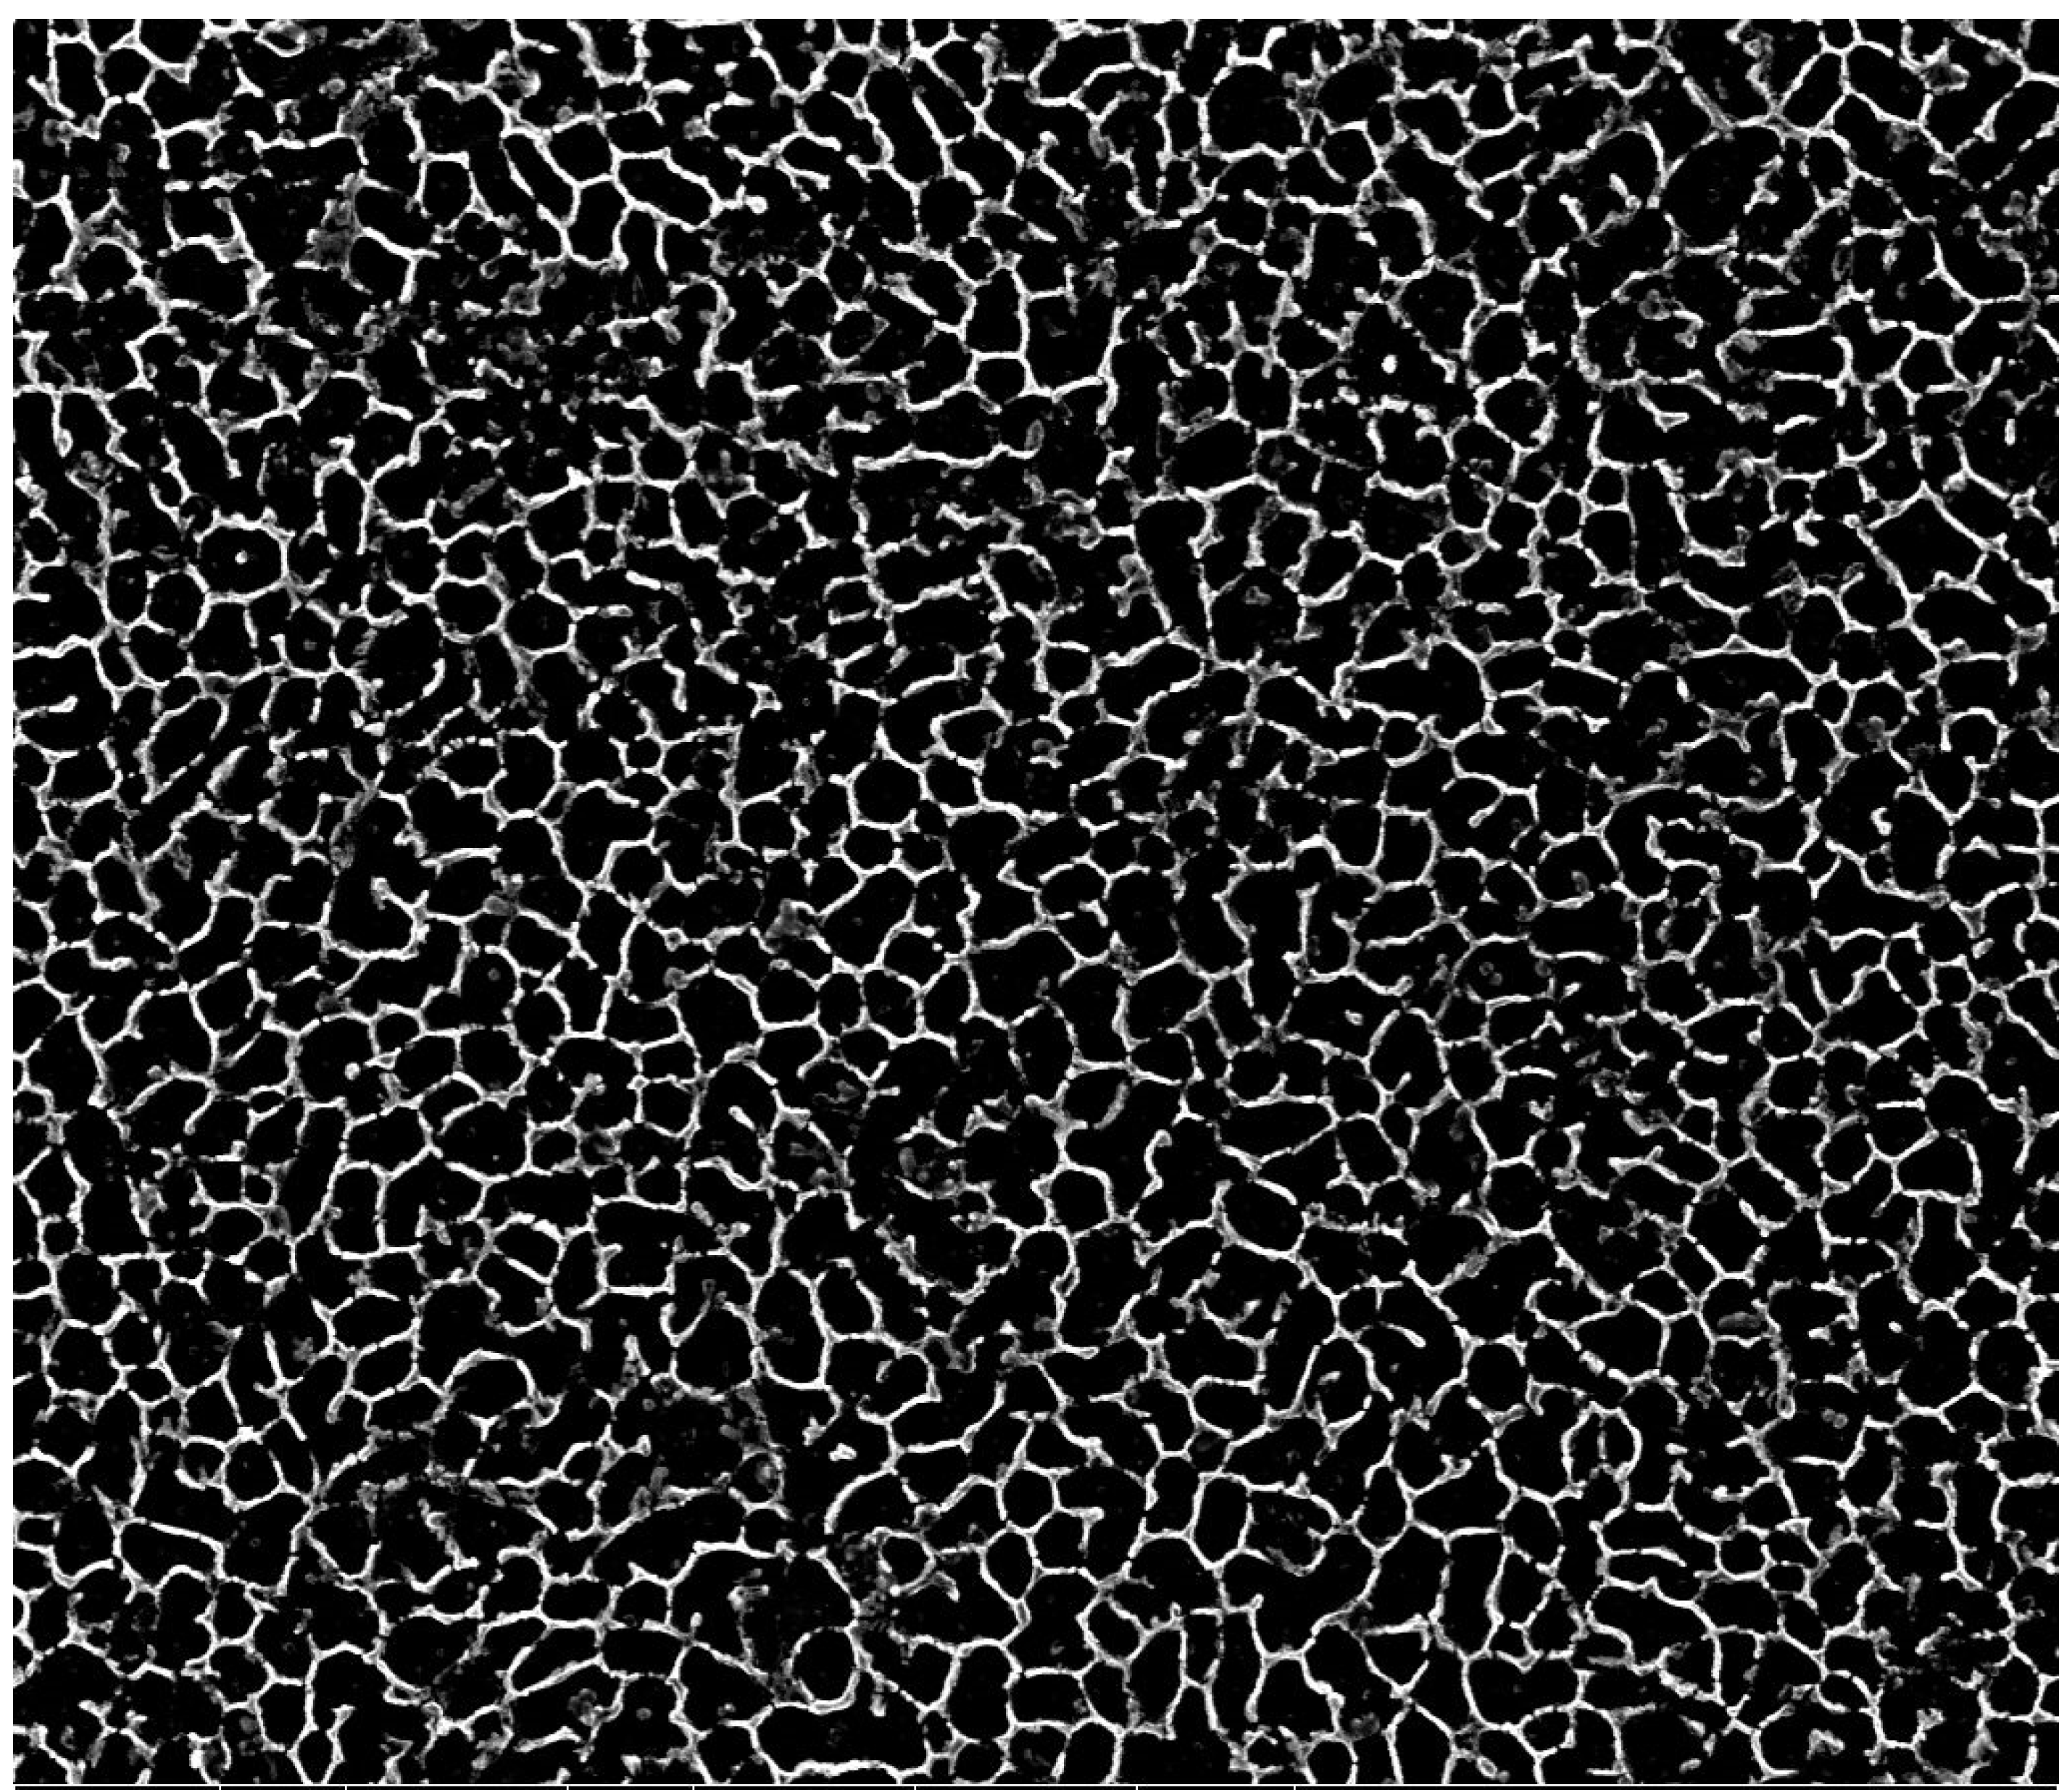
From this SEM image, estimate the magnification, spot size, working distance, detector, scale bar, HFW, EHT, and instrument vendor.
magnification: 25 K X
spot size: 5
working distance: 10.1 mm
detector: ETD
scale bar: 4000 nm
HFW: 11.9 µm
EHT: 20 kV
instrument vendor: FEI